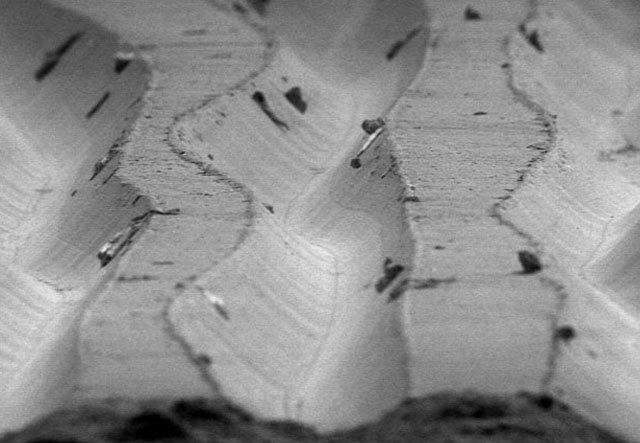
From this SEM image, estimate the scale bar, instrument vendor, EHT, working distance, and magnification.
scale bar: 50000 nm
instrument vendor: other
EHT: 5 kV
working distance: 27 mm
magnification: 0.5 K X